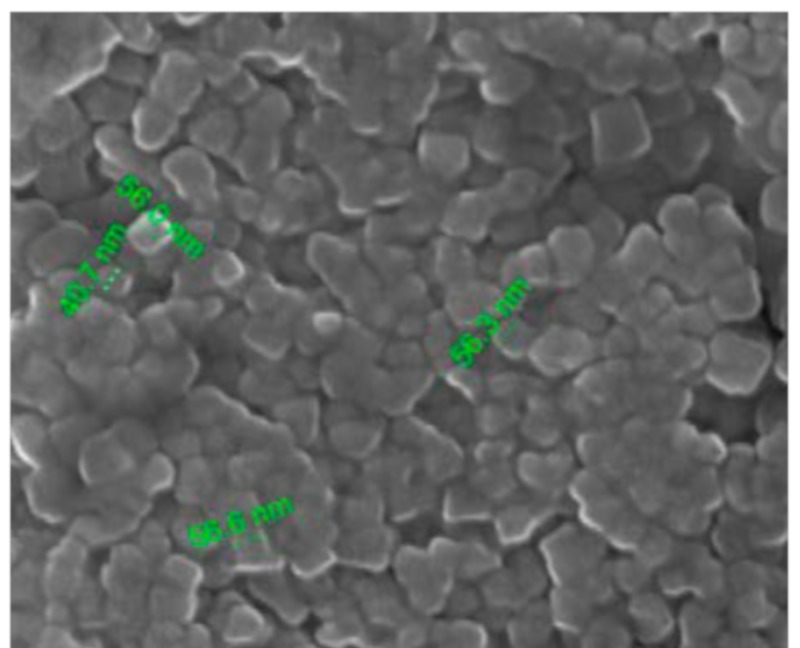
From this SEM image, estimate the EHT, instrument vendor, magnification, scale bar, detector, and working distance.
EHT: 30 kV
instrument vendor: FEI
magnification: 100 K X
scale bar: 500 nm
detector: ETD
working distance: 9.4 mm